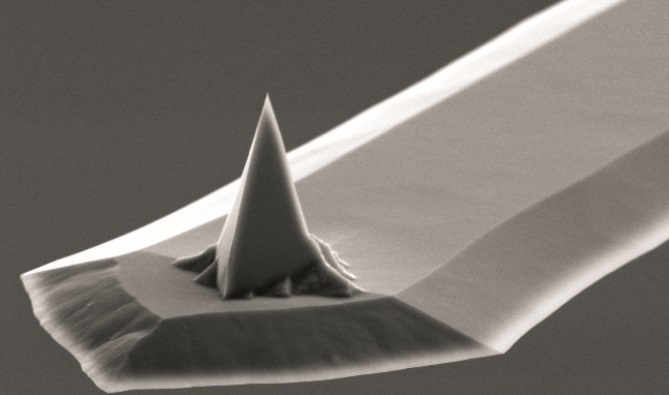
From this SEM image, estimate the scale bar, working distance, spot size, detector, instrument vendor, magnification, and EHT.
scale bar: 10000 nm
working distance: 14.7 mm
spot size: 3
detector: SE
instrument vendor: FEI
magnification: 2.5 K X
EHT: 10 kV